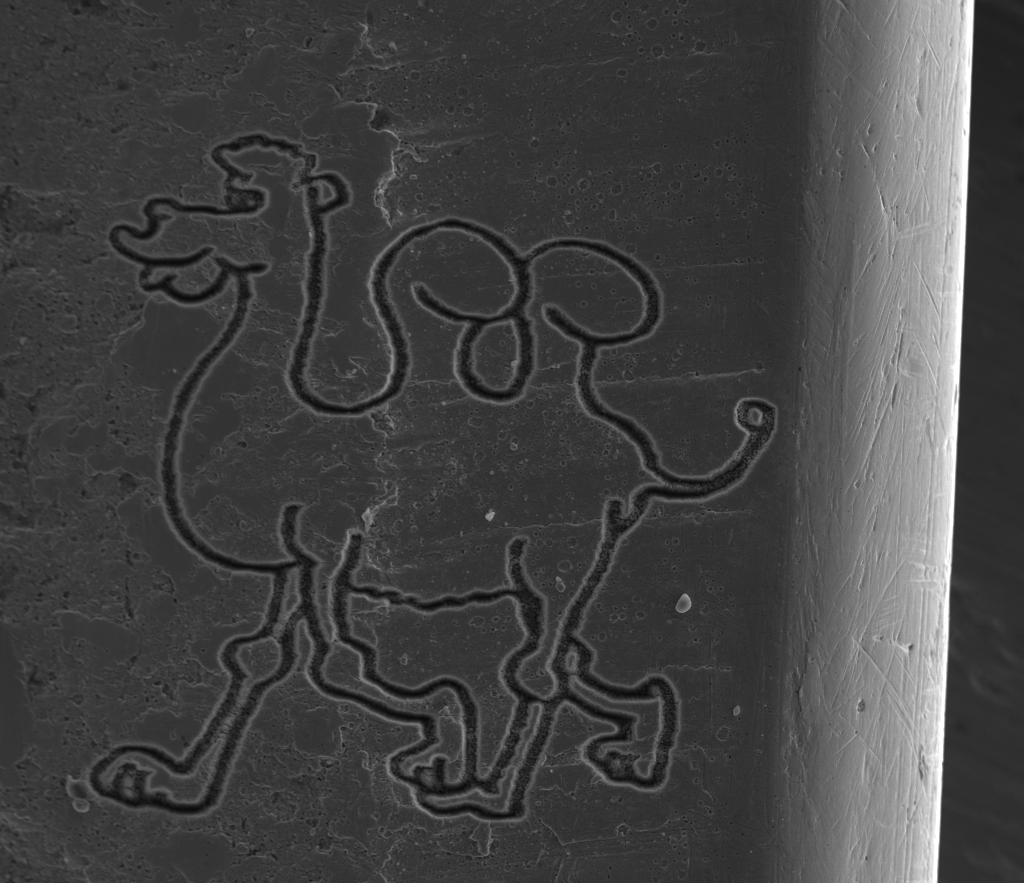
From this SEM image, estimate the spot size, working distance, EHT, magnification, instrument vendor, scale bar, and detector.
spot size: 6.5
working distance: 10 mm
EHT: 15 kV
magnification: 0.5 K X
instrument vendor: FEI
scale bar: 100000 nm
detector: ETD